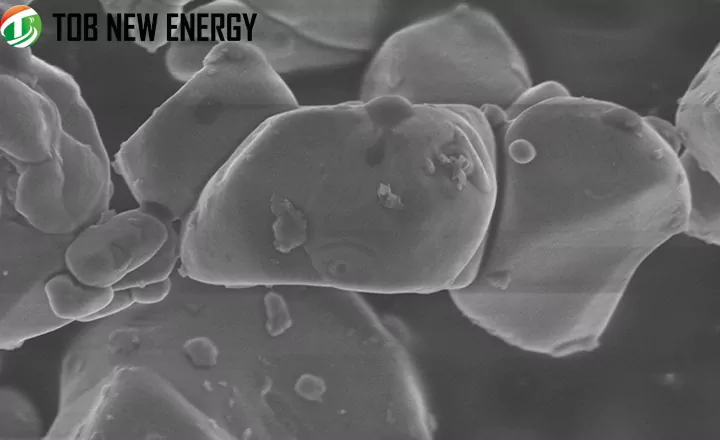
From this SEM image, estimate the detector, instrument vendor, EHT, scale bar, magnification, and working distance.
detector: SE(M)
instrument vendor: Hitachi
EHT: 3 kV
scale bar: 2000 nm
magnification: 20 K X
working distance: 7.8 mm